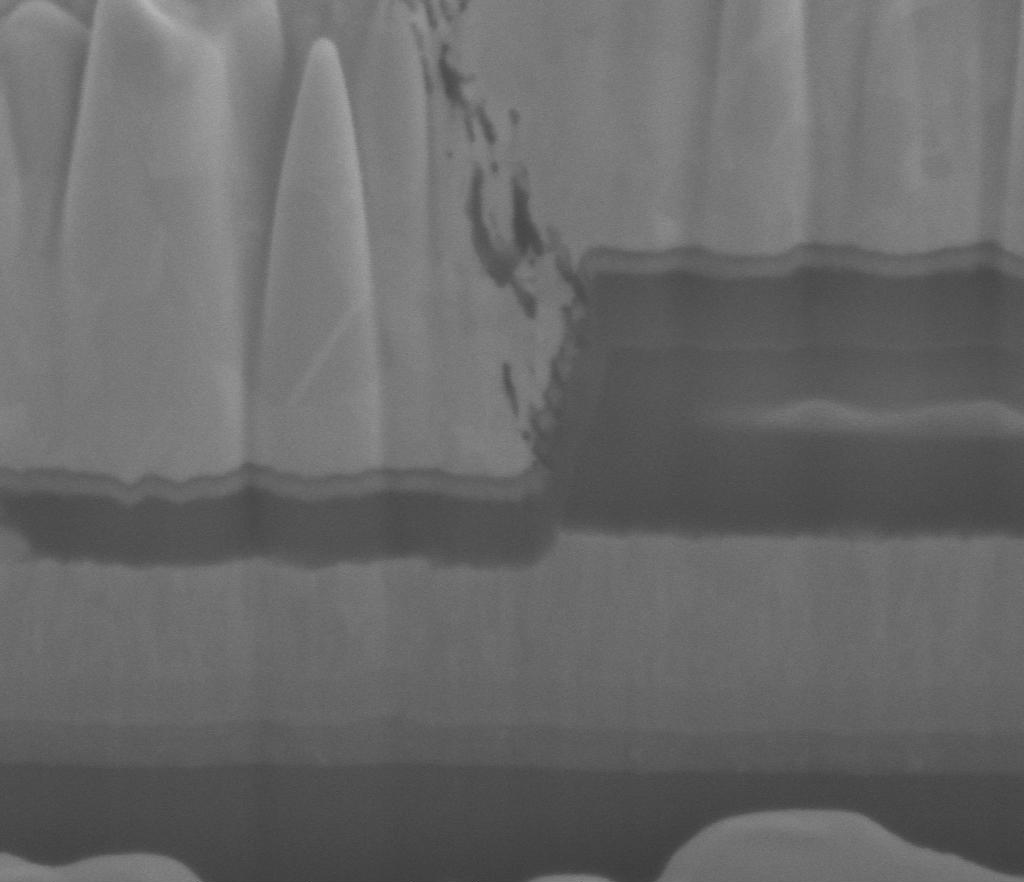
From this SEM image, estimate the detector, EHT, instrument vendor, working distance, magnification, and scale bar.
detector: ETD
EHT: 5 kV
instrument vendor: FEI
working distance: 4.9 mm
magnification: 80 K X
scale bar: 1000 nm